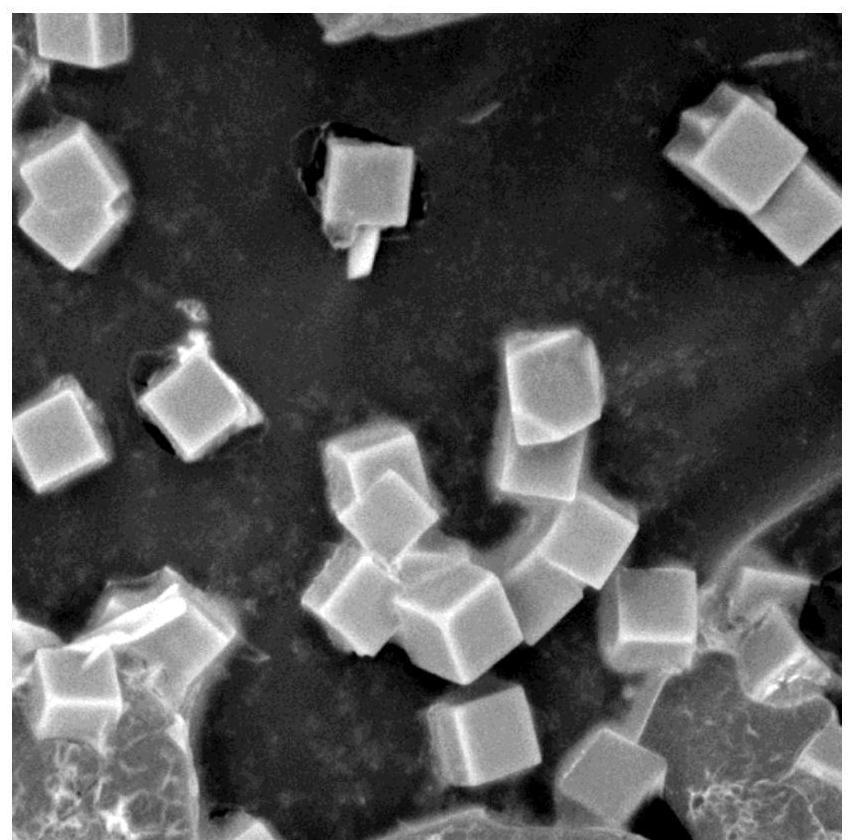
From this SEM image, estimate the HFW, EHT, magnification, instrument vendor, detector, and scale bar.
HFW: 38.4 µm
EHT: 10 kV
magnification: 7 K X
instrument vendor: Thermo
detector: BSD Full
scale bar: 10000 nm